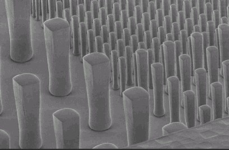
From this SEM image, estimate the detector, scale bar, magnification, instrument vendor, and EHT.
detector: SEI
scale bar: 100000 nm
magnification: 0.16 K X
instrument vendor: JEOL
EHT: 1 kV